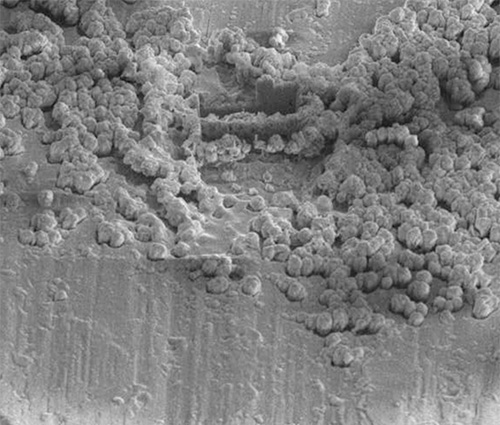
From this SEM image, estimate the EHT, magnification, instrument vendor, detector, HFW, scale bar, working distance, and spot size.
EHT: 10 kV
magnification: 2 K X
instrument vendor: FEI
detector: SED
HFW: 152 µm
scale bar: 20000 nm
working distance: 5 mm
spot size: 3